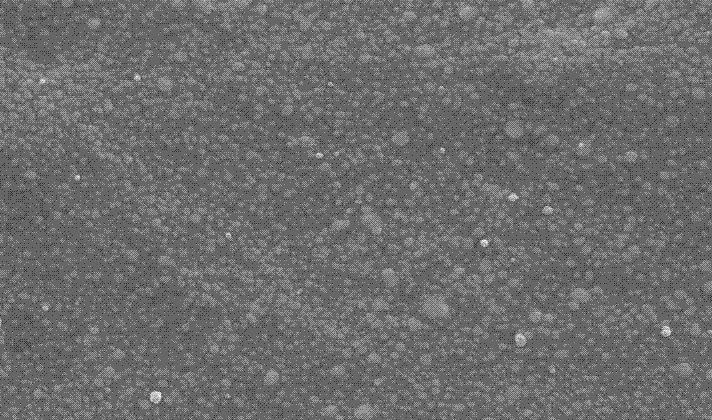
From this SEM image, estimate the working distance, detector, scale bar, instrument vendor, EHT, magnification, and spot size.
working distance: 5.2 mm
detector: SE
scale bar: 2000 nm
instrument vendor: FEI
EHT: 20 kV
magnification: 10 K X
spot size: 3